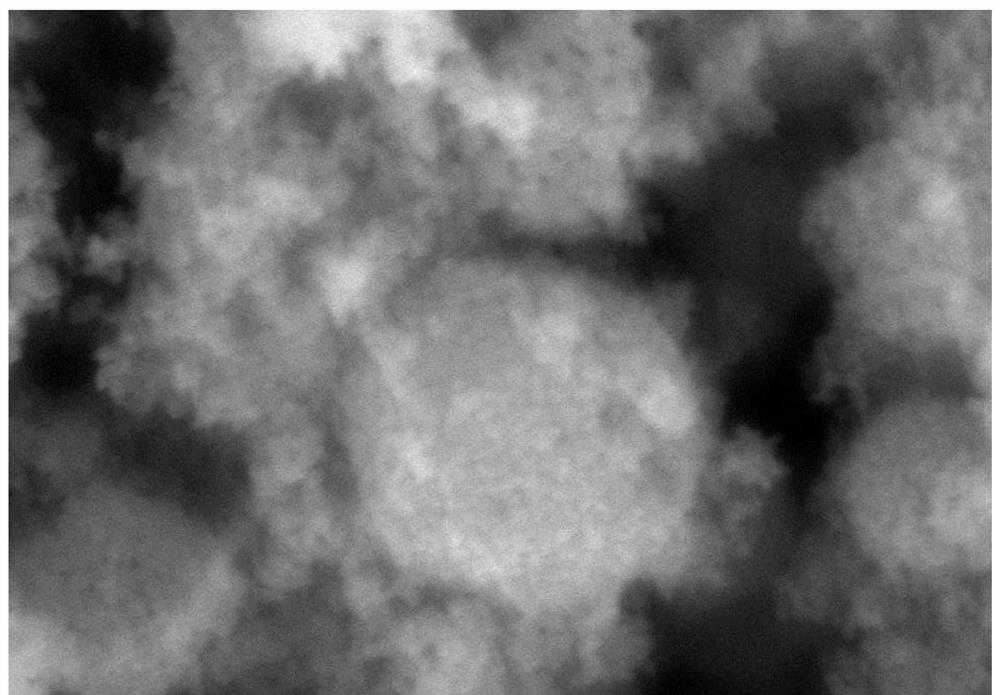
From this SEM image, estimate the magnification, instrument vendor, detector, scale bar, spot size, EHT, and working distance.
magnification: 20 K X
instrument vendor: JEOL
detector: SEI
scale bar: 1000 nm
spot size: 30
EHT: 25 kV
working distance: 10 mm